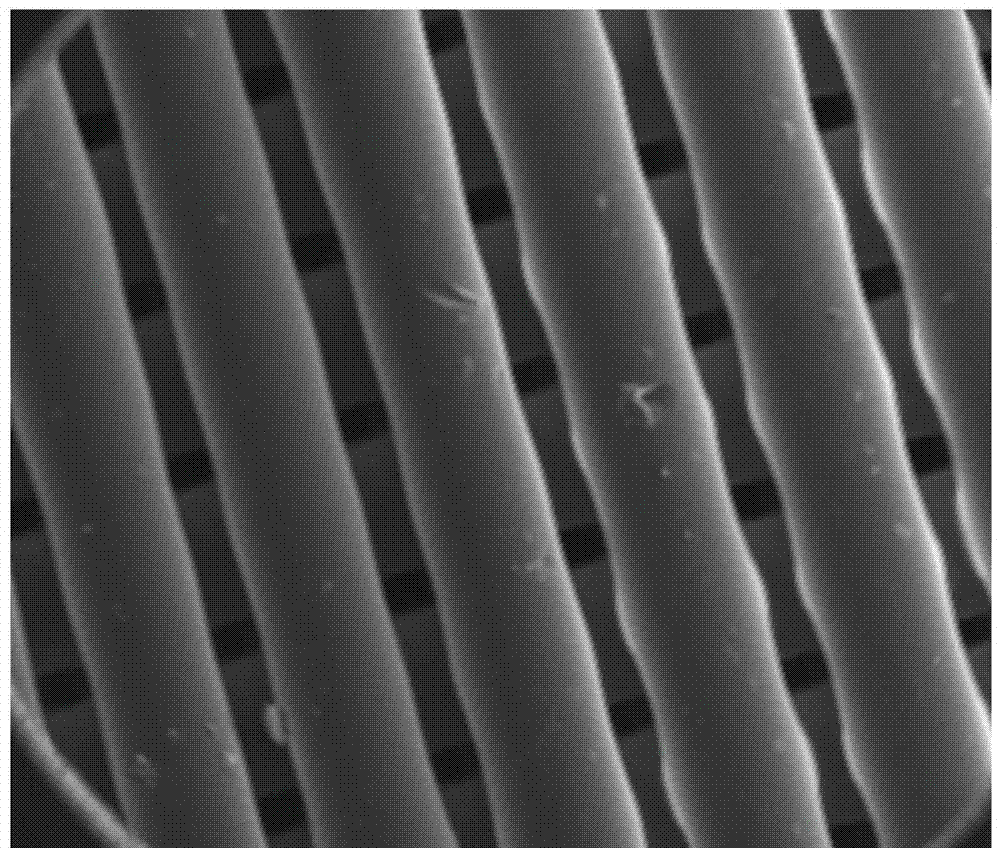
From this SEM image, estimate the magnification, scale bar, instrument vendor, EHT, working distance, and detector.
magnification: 0.15 K X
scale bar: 500000 nm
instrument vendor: FEI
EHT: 15 kV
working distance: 5 mm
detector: ETD